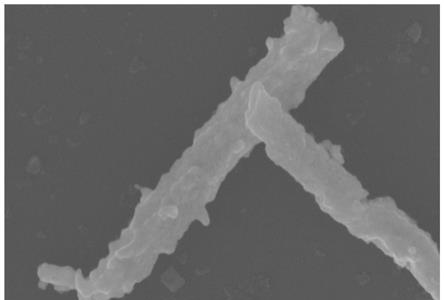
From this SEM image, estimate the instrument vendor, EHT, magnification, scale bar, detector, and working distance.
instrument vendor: Zeiss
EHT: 10 kV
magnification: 50 K X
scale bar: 200 nm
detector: InLens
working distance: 7.1 mm